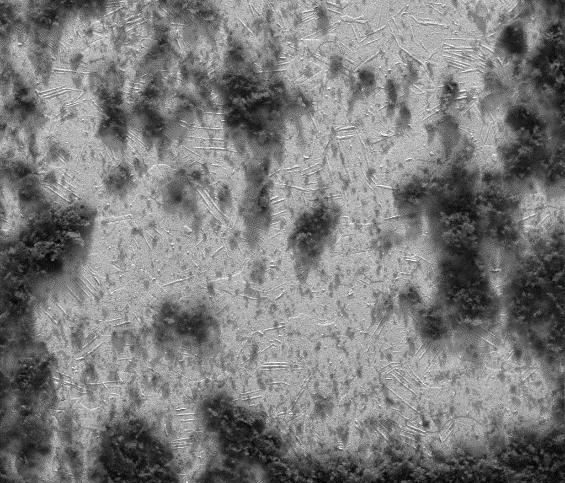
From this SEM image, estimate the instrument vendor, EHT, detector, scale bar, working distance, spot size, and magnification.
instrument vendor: FEI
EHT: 30 kV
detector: Mix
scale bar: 500000 nm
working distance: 9.6 mm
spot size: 5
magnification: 0.1 K X